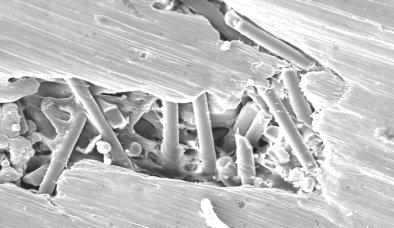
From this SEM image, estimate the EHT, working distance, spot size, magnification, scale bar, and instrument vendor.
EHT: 20 kV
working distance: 10 mm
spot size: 5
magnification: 0.4 K X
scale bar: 50000 nm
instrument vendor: FEI